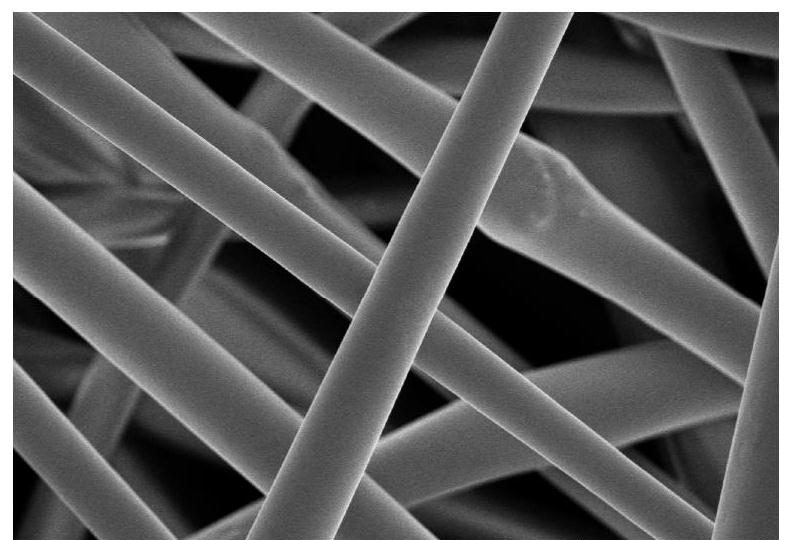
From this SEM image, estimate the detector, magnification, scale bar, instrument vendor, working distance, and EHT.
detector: SE(UL)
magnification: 3 K X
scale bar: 10000 nm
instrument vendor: Hitachi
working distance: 8.5 mm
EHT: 3 kV